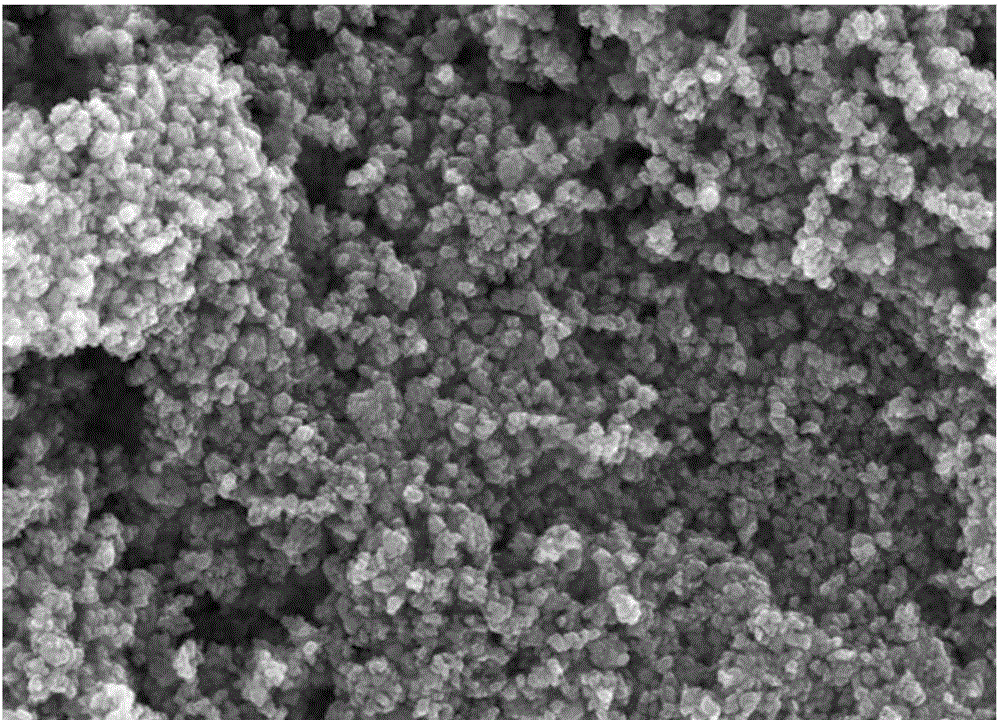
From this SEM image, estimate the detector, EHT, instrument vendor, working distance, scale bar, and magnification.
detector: InLens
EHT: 15 kV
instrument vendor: Zeiss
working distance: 6.3 mm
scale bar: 200 nm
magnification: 50 K X